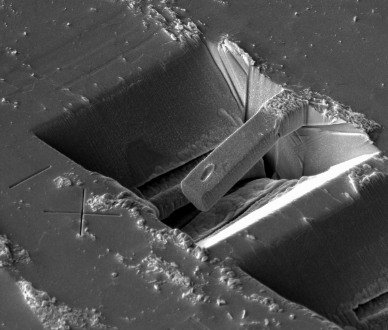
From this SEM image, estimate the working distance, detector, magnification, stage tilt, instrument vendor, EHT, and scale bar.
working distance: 4.2 mm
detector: ICE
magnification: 3 K X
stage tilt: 52°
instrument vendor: FEI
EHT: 10 kV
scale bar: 10000 nm